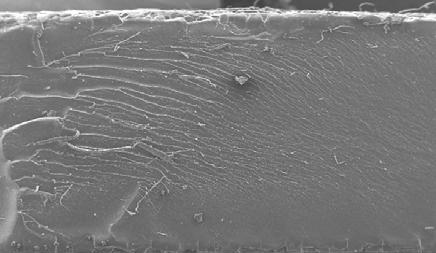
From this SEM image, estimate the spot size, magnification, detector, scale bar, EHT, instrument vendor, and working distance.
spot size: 5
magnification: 0.05 K X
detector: SE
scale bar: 1000000 nm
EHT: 20 kV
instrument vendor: FEI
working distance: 10.2 mm